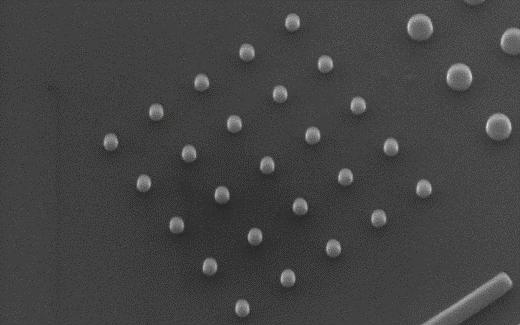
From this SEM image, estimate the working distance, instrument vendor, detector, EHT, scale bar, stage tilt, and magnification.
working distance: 17.6 mm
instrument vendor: Zeiss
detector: SE2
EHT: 10 kV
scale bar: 100 nm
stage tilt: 23.3°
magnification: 120 K X